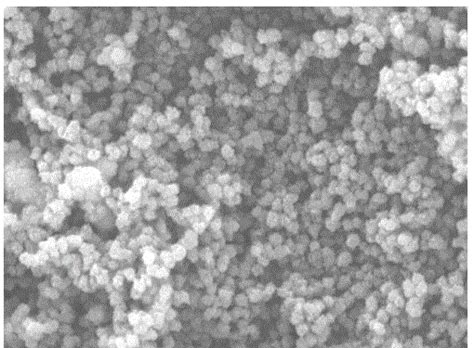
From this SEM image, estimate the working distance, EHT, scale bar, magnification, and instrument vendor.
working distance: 14.11 mm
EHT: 15 kV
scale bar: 2000 nm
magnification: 20 K X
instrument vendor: Tescan